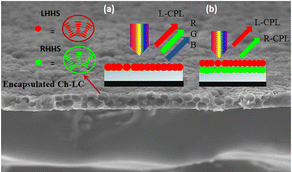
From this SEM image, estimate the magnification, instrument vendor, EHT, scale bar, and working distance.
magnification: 0.5 K X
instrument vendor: Hitachi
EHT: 20 kV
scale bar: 100000 nm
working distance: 13.7 mm